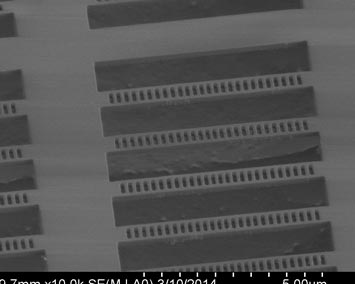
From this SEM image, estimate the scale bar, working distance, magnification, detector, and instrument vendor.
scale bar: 5000 nm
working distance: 9.7 mm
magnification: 10 K X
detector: SE(M,LA0)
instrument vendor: Hitachi